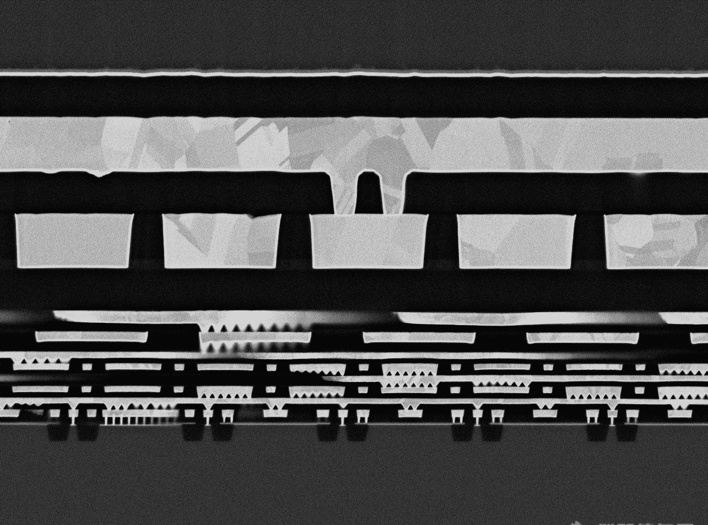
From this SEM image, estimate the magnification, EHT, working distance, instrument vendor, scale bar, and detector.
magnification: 10 K X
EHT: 5 kV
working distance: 8 mm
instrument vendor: JEOL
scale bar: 1000 nm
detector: LABE